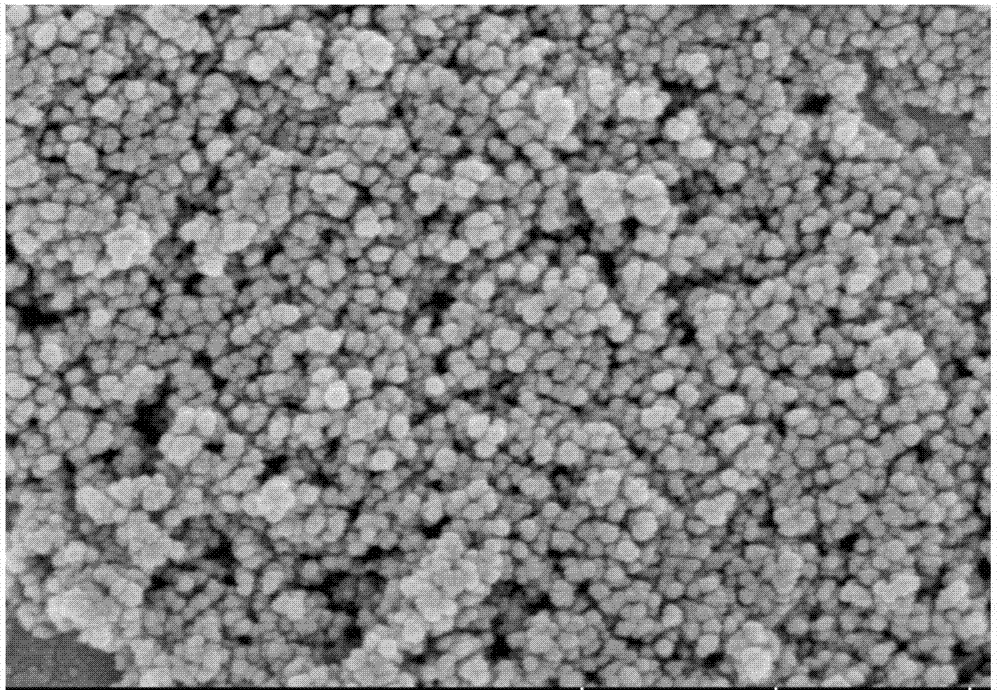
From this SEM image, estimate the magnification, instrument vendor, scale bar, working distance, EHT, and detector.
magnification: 100 K X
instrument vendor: Hitachi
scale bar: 500 nm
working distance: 6.7 mm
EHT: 3 kV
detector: SE(UL)